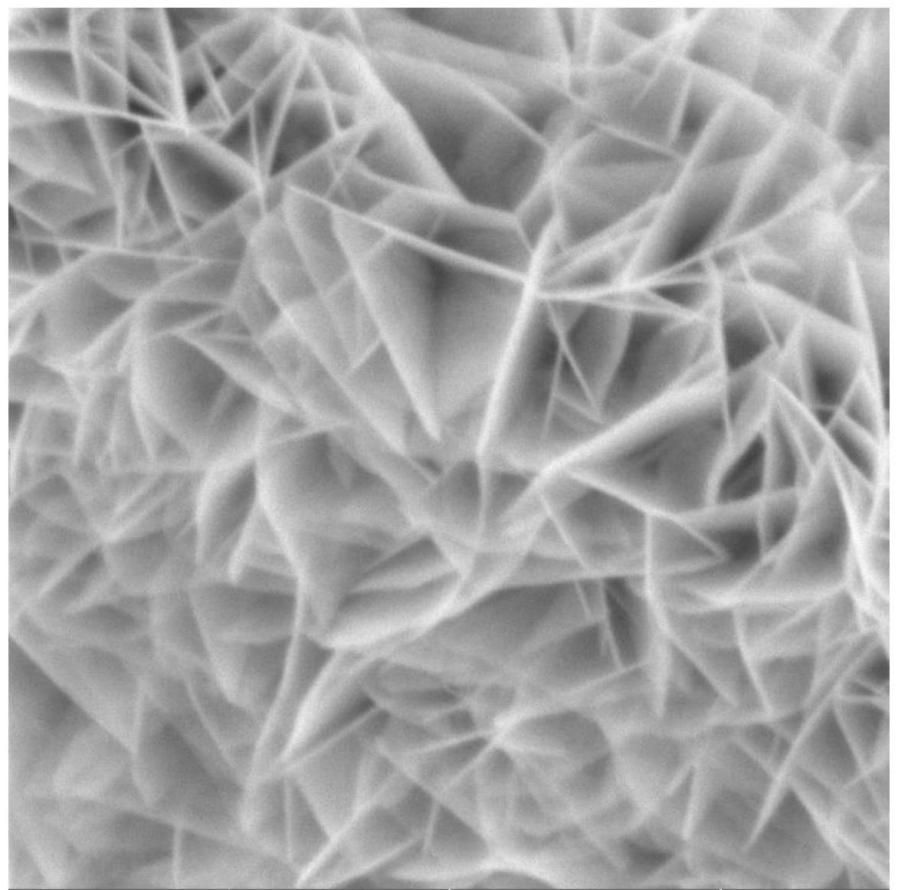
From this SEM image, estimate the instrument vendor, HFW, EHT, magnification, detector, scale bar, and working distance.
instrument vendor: Tescan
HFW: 6.5 µm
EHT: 10 kV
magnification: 42.6 K X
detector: SE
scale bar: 2000 nm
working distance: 16.6 mm